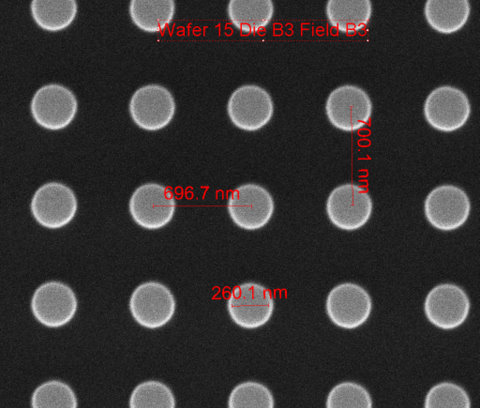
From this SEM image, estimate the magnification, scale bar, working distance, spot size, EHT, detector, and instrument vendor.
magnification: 75 K X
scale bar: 1000 nm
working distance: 10.2 mm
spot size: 1.5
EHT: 10 kV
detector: ETD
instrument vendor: FEI